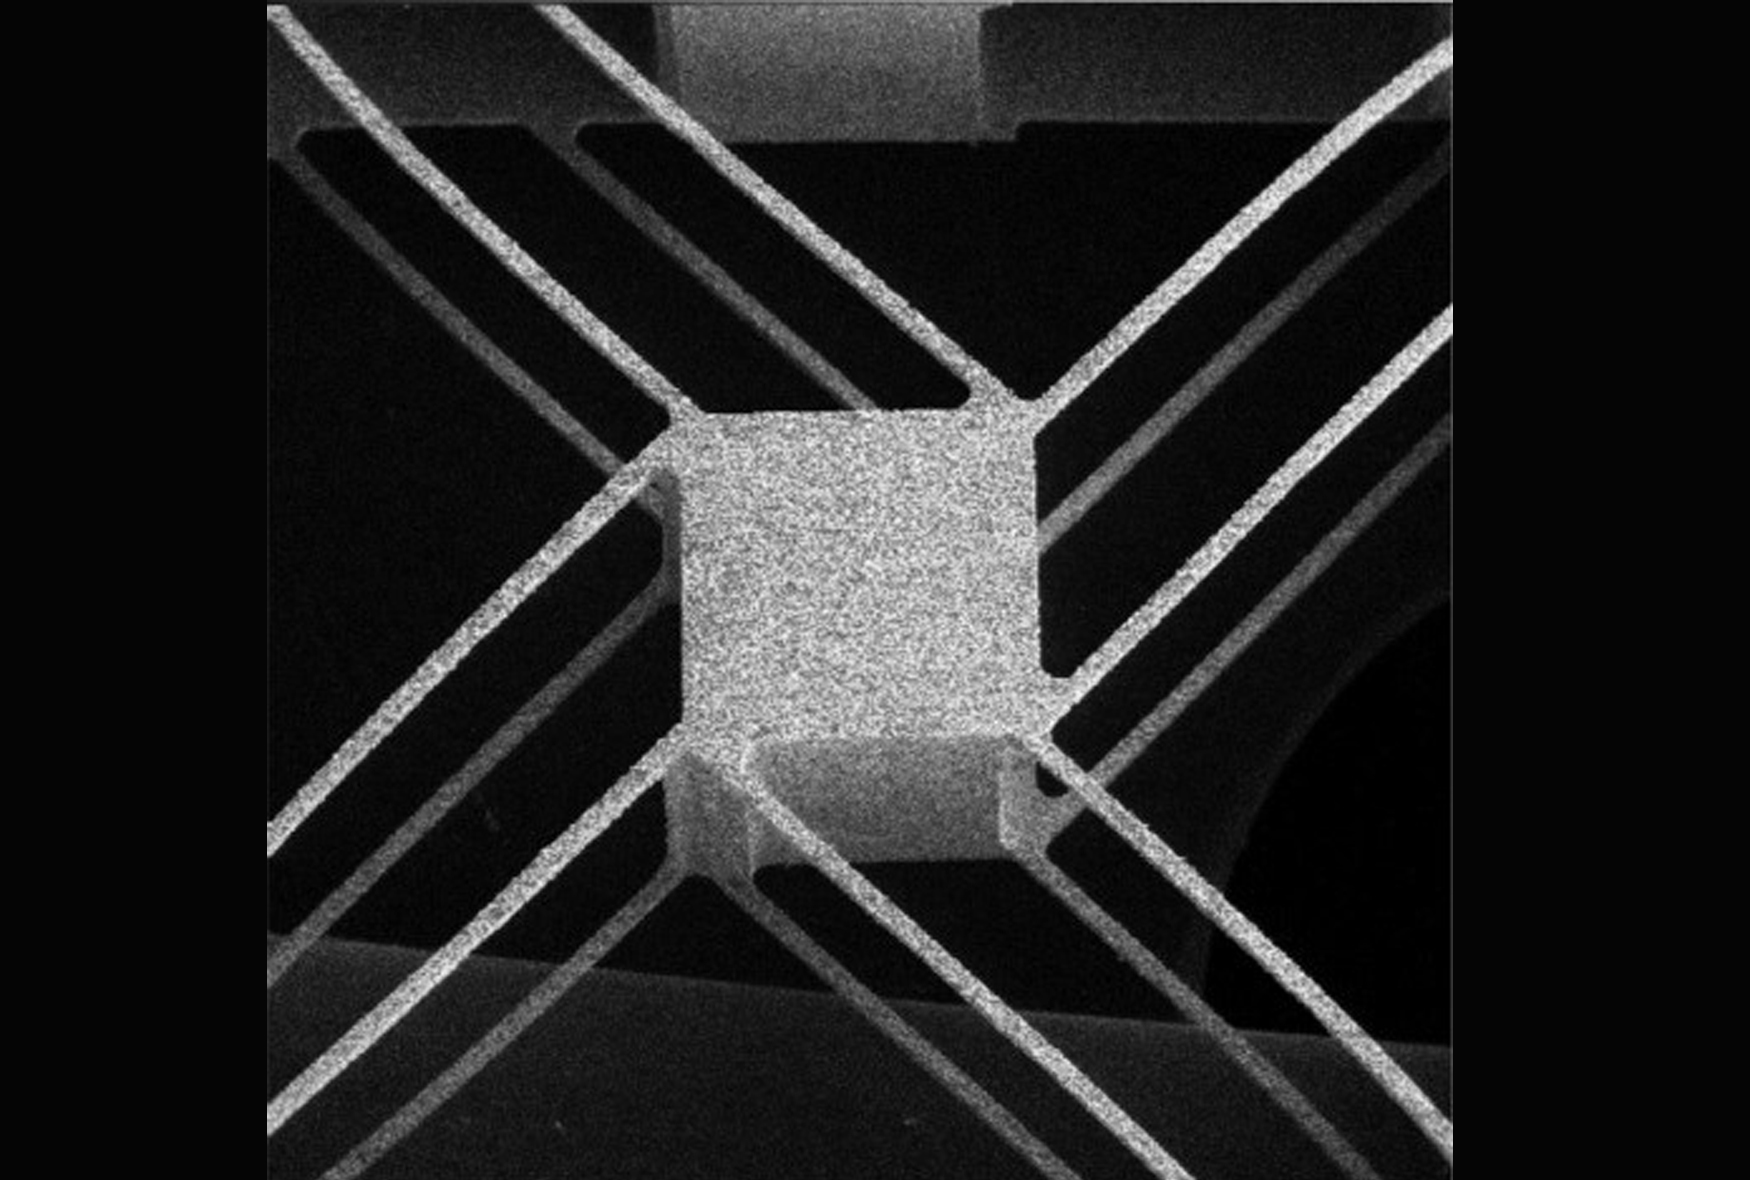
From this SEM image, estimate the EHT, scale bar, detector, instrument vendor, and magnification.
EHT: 30 kV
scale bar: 1000000 nm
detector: SE Detector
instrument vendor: Tescan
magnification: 0.059 K X